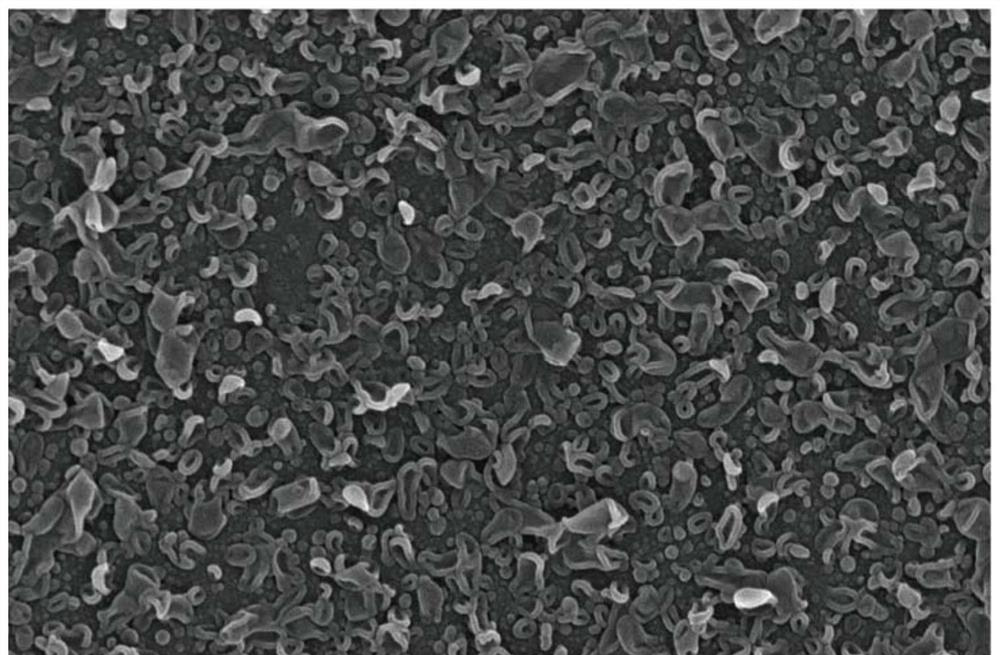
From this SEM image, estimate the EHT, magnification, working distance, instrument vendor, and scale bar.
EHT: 15 kV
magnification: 80 K X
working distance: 5.9 mm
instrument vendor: FEI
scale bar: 2000 nm